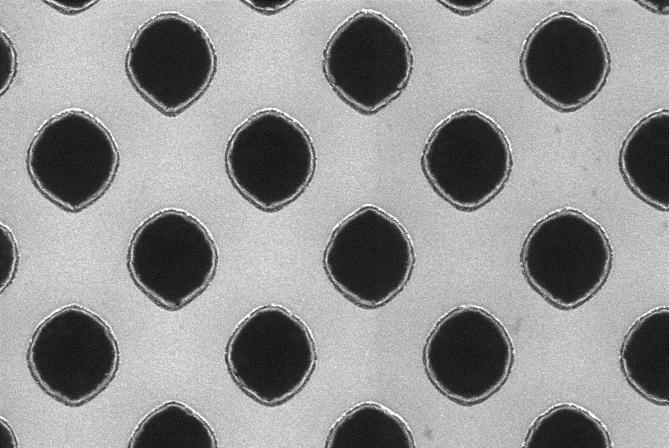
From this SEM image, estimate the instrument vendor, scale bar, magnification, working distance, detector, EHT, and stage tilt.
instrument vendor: Zeiss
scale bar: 100 nm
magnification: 100 K X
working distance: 3.5 mm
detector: InLens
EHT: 5 kV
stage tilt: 0°